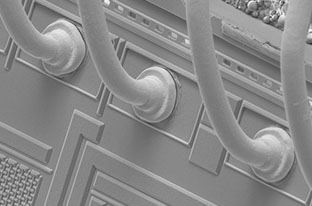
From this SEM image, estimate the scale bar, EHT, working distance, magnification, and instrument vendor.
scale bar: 100000 nm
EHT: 10 kV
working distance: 5.8 mm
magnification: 1.2 K X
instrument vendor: FEI